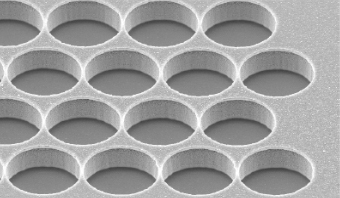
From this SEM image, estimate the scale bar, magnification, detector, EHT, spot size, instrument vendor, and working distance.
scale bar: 20000 nm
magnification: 3 K X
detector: SE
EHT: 10 kV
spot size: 3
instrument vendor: FEI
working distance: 24 mm